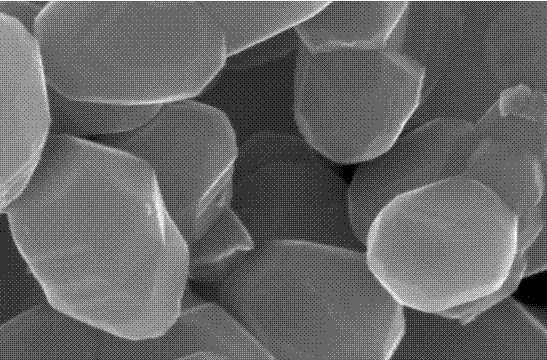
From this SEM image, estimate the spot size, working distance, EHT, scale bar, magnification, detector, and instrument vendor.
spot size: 3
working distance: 5.2 mm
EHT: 15 kV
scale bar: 300 nm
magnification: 400 K X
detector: TLD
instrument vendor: FEI